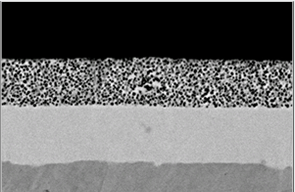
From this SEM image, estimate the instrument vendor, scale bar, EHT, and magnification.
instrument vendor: JEOL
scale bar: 5000 nm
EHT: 15 kV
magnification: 5 K X